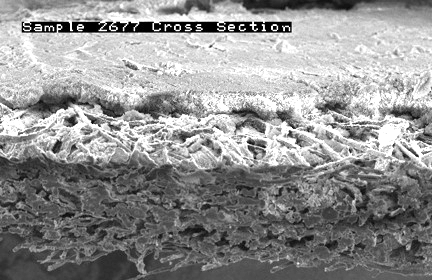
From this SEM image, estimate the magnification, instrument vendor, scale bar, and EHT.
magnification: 0.1 K X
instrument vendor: JEOL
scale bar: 100000 nm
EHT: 15 kV